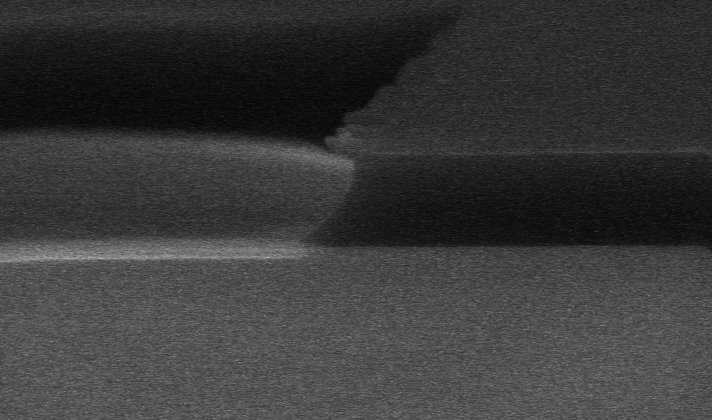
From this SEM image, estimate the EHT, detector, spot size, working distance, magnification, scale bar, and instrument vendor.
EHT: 18 kV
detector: TLD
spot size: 3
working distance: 4.7 mm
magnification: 37.733 K X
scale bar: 2000 nm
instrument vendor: FEI